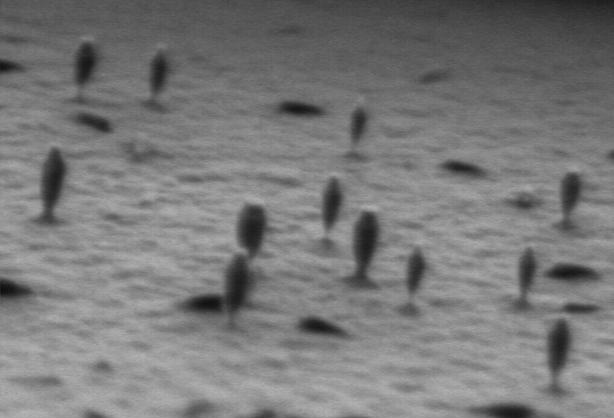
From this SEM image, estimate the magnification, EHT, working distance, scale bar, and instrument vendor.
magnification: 50 K X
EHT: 1 kV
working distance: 4 mm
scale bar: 500 nm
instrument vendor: Hitachi